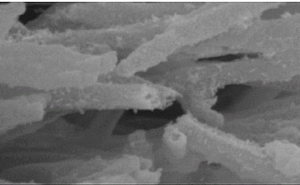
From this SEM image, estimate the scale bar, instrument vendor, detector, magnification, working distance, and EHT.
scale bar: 500 nm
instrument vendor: Hitachi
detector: SE(M)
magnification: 100 K X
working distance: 14.7 mm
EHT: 5 kV